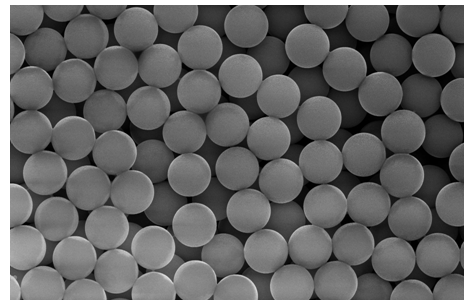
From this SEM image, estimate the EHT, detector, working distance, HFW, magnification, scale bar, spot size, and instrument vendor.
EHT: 30 kV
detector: SE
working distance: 10.4 mm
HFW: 82.9 µm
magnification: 5 K X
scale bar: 30000 nm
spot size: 4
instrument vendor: FEI